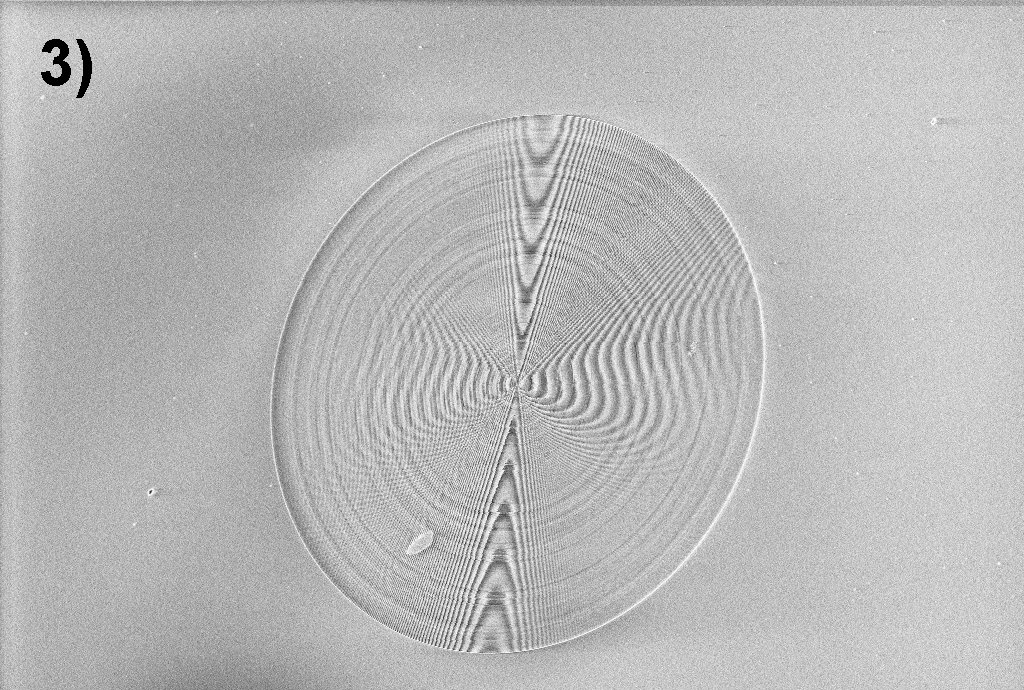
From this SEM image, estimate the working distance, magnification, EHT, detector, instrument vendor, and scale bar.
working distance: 5.1 mm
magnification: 0.309 K X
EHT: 5 kV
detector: InLens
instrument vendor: Zeiss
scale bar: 20000 nm